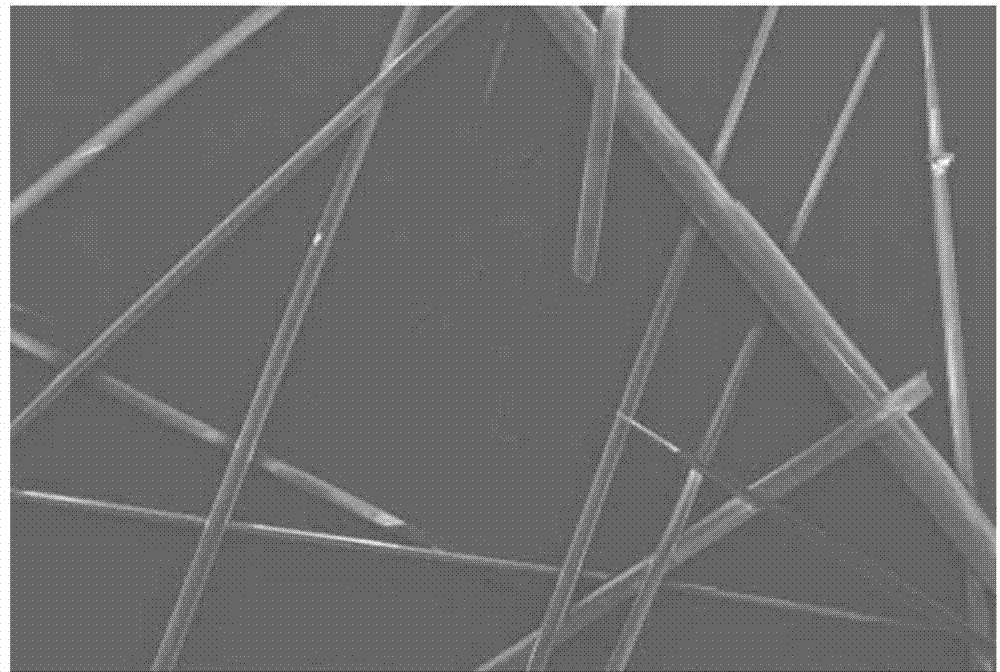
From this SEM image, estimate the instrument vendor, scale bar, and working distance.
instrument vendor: Hitachi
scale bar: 1000000 nm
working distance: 11.8 mm